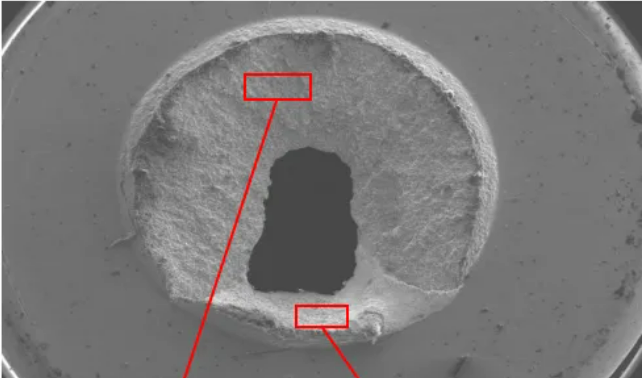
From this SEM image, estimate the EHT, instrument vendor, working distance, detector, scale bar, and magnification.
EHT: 15 kV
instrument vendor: Hitachi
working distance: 14.9 mm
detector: LM(L)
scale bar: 2000000 nm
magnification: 0.025 K X